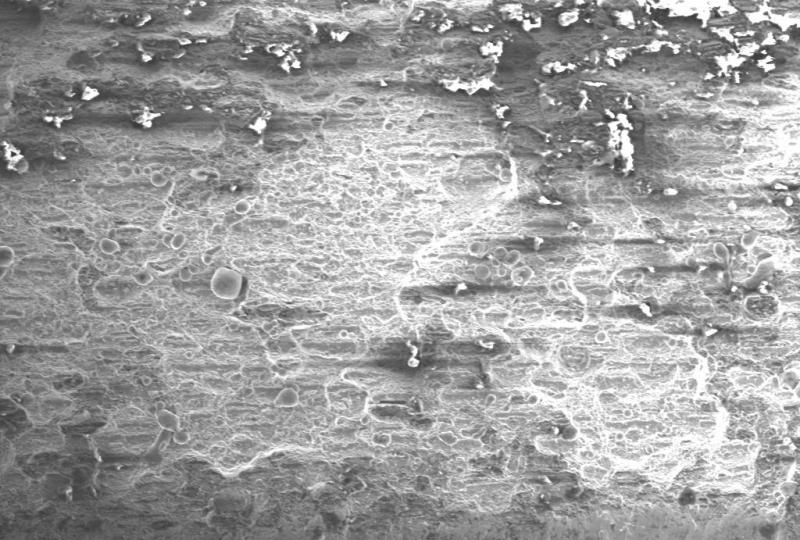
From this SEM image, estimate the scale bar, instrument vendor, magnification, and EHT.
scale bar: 1000000 nm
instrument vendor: other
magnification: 0.1 K X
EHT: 20 kV